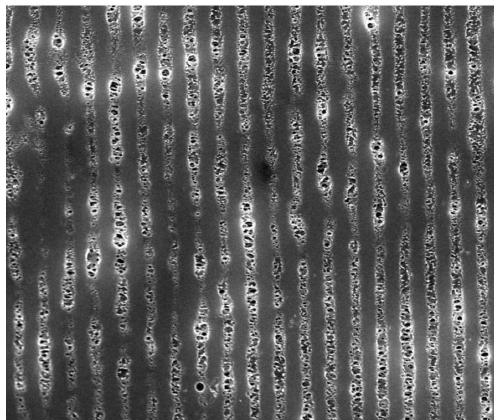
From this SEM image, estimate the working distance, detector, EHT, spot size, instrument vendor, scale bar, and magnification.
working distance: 5.9 mm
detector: TLD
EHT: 10 kV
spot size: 3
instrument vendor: FEI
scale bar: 5000 nm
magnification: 20 K X